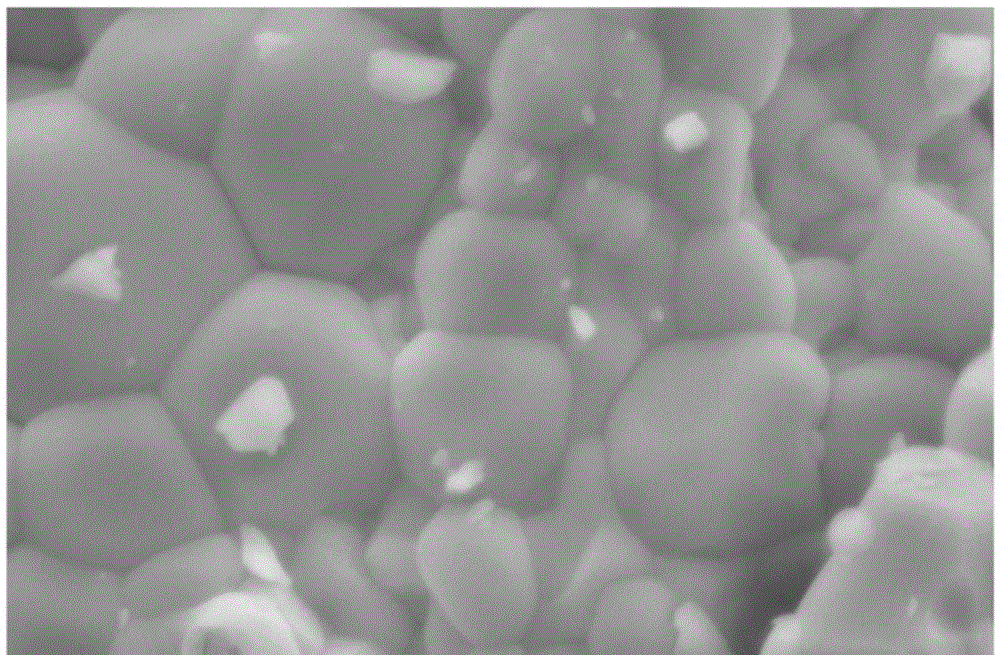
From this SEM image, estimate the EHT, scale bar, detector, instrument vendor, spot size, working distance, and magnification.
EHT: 10 kV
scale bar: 2000 nm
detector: SE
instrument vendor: FEI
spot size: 4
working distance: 10.8 mm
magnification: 20 K X